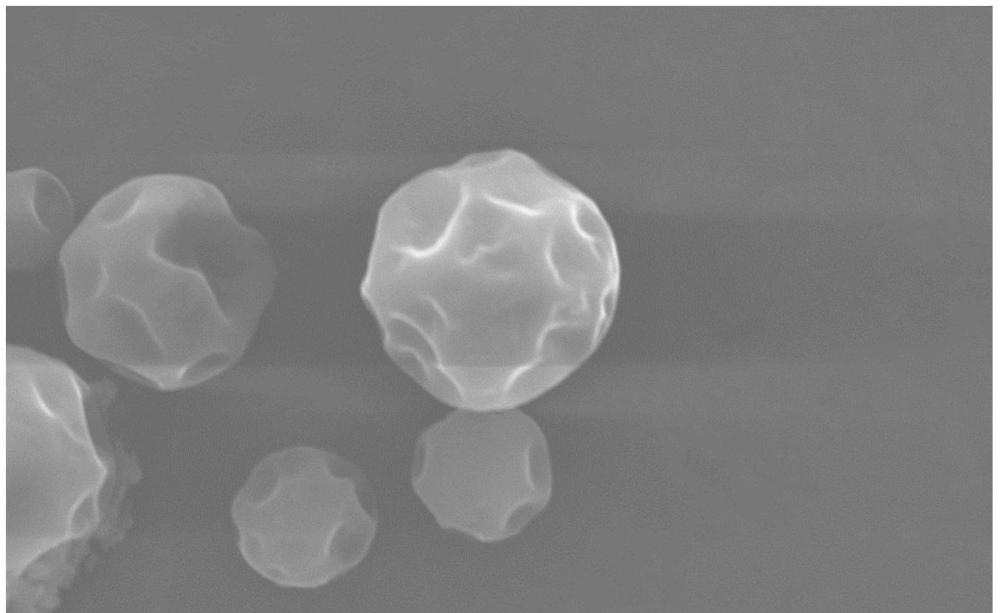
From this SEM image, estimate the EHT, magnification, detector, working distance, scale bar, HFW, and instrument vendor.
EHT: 15 kV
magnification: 15 K X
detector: SED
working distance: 9.398 mm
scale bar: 10000 nm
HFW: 34.6 µm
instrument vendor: Thermo